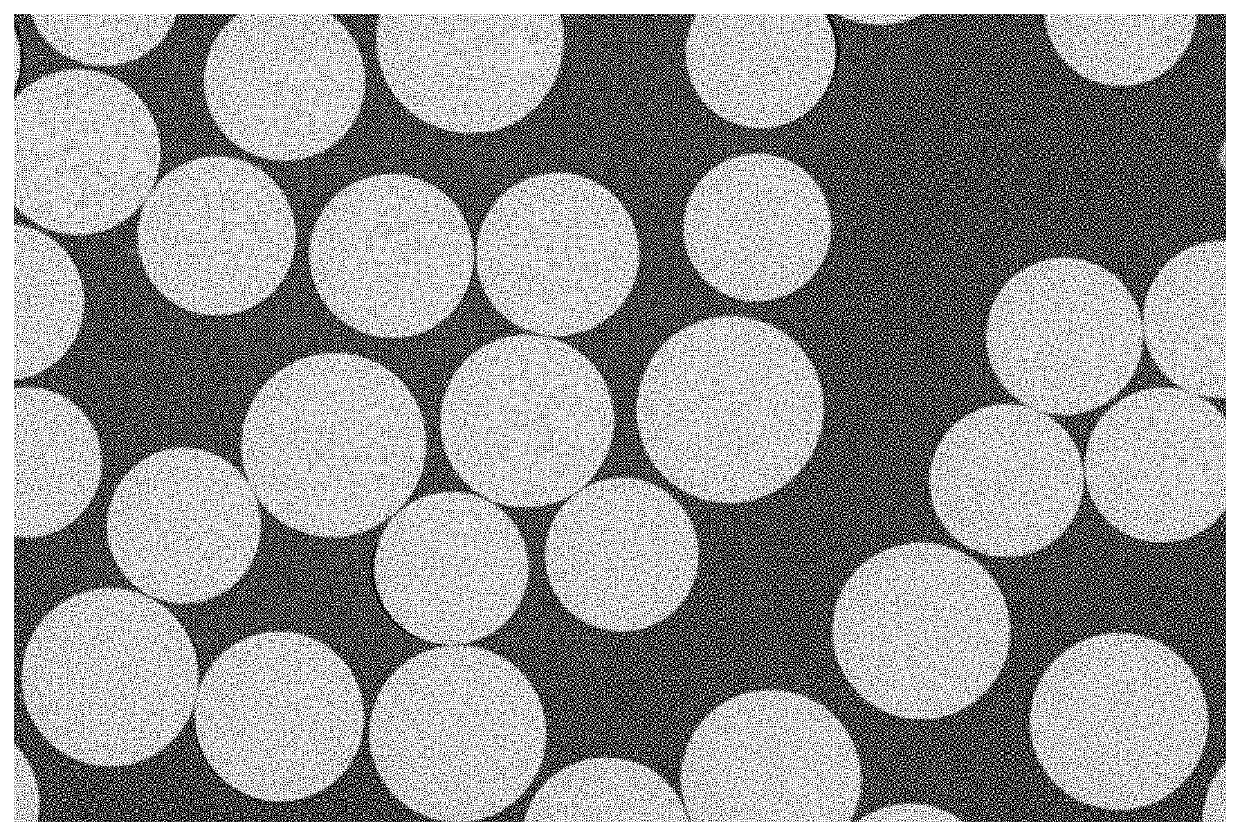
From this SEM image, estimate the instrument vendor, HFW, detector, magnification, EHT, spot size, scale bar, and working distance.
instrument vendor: FEI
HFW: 127 µm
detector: vCD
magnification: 1 K X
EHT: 10 kV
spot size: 4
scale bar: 30000 nm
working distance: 10 mm